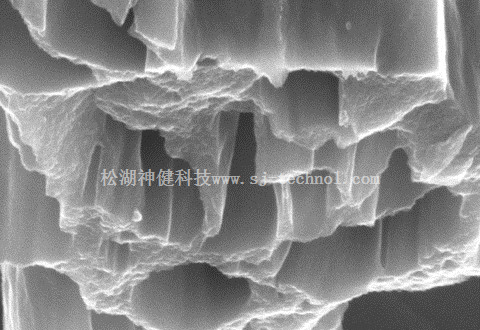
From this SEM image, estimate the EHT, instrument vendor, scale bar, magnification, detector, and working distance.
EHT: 5 kV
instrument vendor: Hitachi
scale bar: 1000 nm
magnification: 50 K X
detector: SE(U)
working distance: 8.6 mm